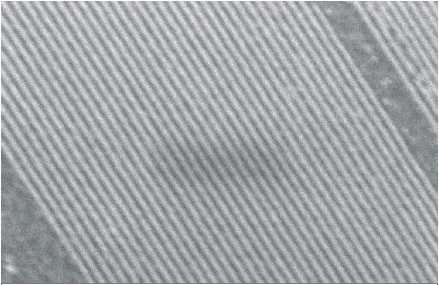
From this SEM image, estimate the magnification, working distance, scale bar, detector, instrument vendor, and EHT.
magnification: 86.53 K X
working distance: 10 mm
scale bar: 200 nm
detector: InLens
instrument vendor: Zeiss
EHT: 5 kV